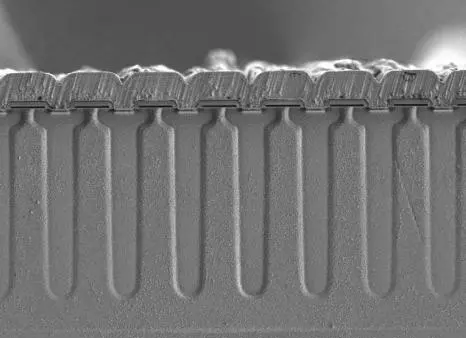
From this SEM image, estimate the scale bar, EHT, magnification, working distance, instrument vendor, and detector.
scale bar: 40000 nm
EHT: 1 kV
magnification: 1.3 K X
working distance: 10.2 mm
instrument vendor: Hitachi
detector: SE(L)